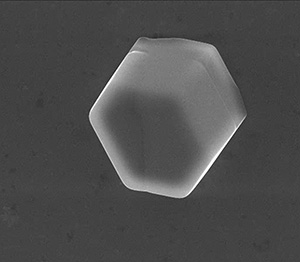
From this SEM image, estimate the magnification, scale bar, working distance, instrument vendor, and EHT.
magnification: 2.995 K X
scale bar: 30000 nm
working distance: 10.8 mm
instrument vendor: FEI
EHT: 20 kV